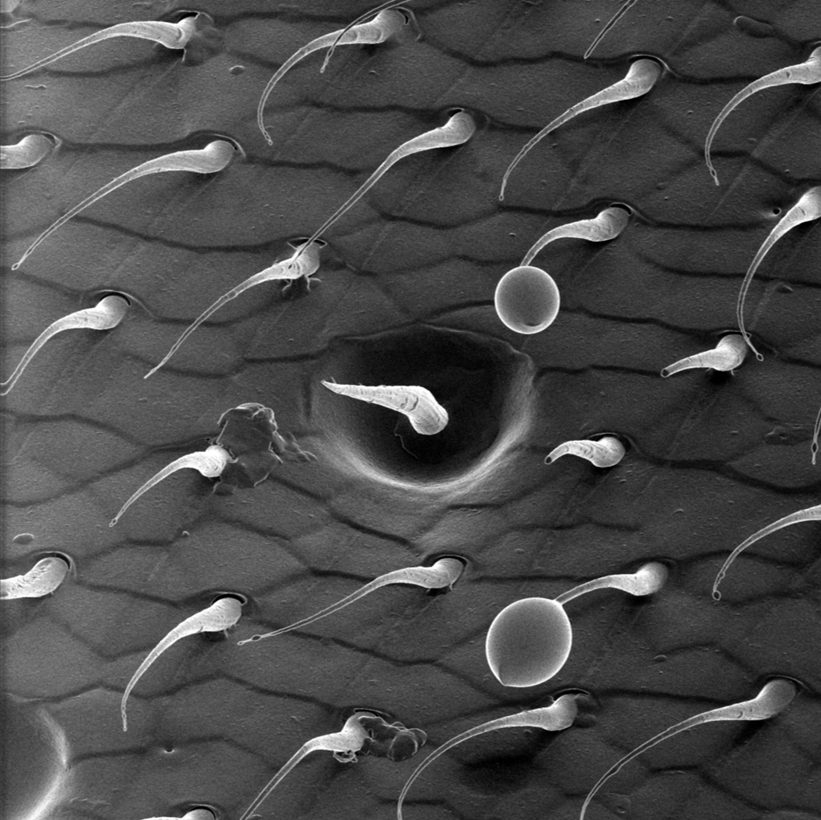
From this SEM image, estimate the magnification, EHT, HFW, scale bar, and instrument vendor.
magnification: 2.40429 K X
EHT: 30 kV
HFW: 80 µm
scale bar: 10000 nm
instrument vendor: Zeiss